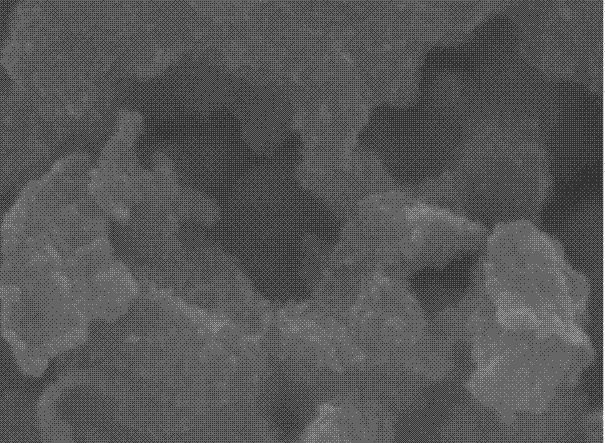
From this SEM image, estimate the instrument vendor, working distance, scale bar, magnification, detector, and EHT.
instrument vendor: JEOL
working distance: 10 mm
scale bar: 100 nm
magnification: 110 K X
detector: LED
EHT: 15 kV